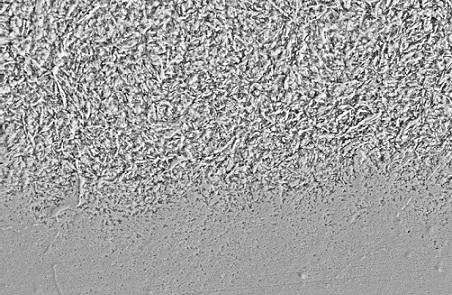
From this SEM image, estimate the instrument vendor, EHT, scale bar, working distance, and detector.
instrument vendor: Zeiss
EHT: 20 kV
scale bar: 10000 nm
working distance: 12 mm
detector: SE2